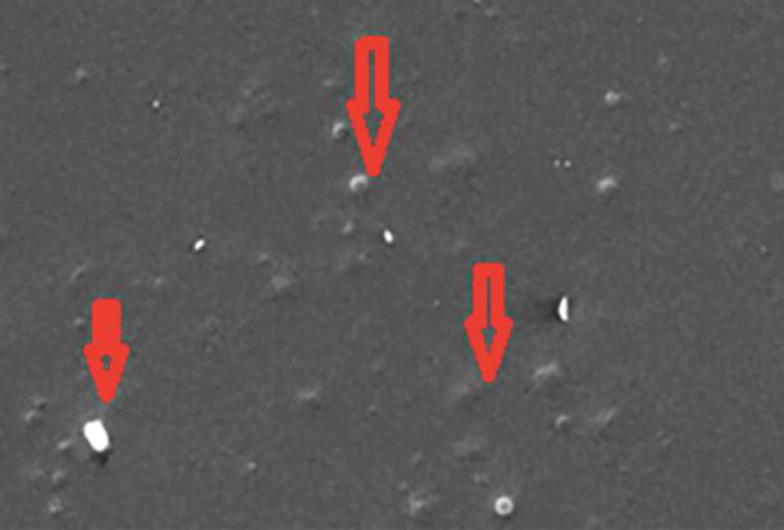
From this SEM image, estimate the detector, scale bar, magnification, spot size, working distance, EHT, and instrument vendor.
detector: SE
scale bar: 20000 nm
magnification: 0.625 K X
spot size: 5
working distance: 13.6 mm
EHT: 25 kV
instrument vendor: FEI